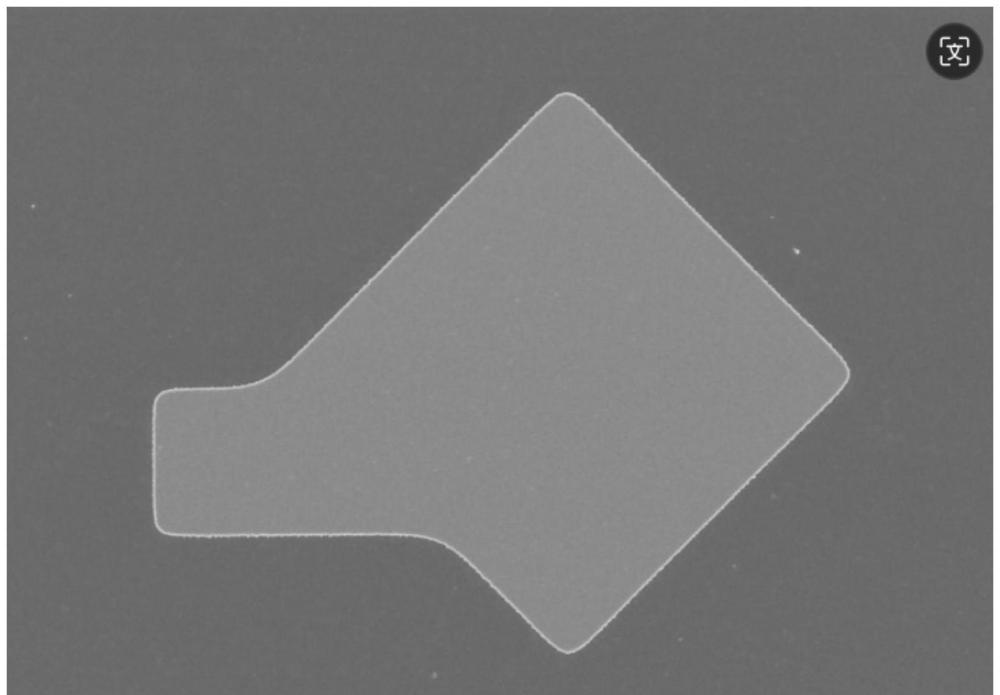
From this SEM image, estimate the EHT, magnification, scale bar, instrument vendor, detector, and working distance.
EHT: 5 kV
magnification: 2.5 K X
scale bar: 20000 nm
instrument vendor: Hitachi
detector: SE(U)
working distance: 11.4 mm